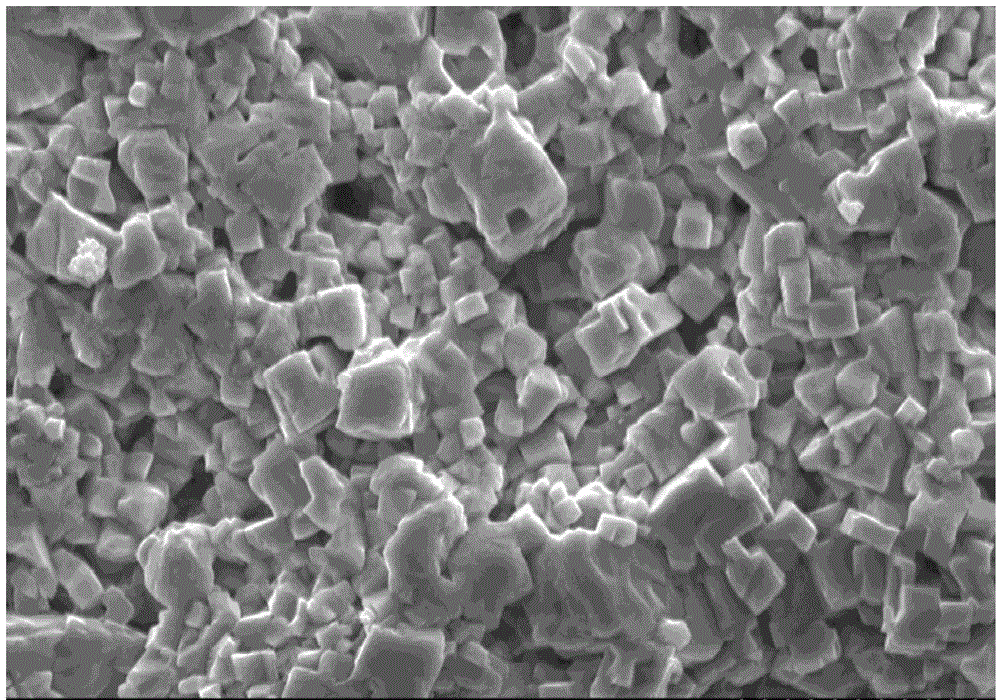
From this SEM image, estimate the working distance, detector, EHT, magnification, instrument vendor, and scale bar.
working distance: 31.5 mm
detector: SE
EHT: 15 kV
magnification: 3 K X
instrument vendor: Hitachi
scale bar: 10000 nm